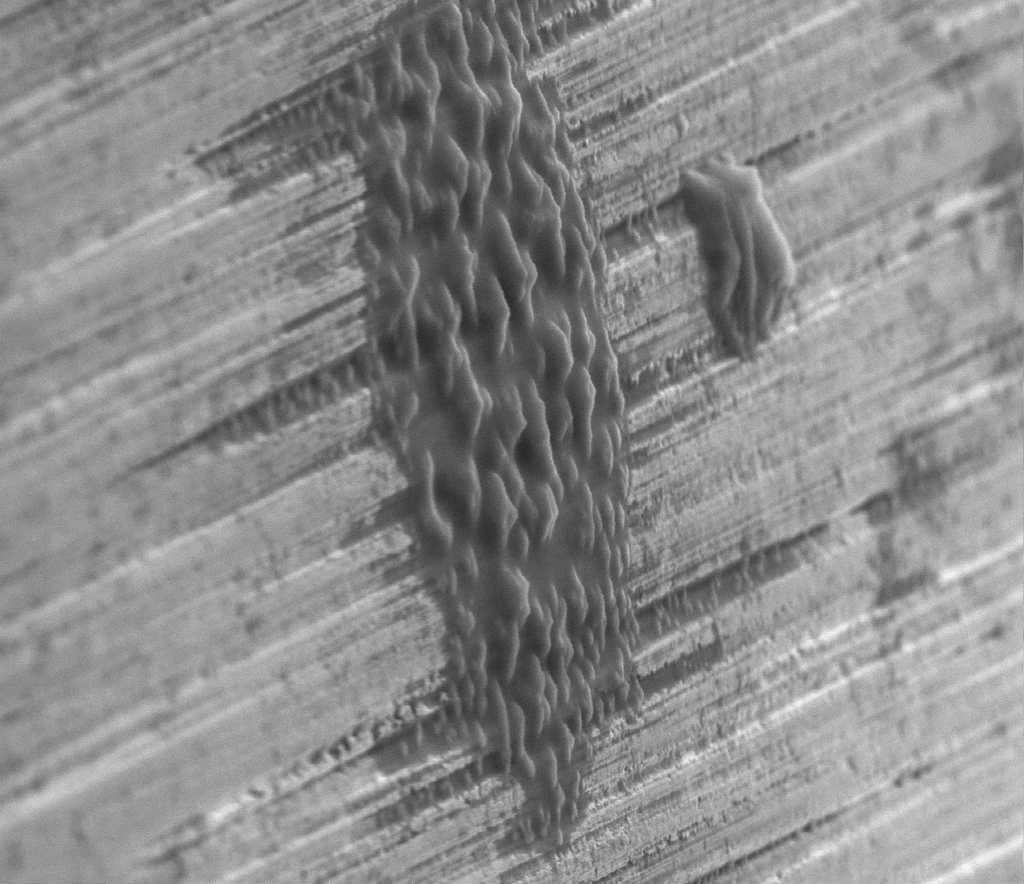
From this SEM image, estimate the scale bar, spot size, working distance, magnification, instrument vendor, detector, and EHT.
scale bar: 50000 nm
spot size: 4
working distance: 12.4 mm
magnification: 1 K X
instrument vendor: FEI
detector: ETD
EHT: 30 kV